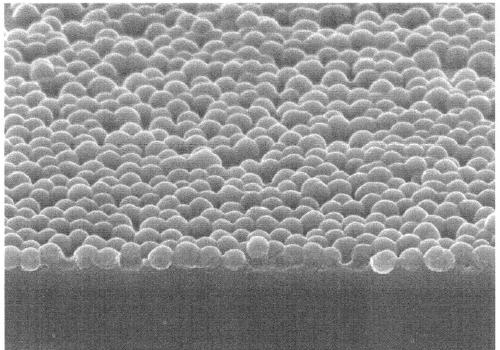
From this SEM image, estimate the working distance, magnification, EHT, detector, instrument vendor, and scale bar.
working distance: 11.7 mm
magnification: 50 K X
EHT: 5 kV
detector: SE(U)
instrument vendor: Hitachi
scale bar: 1000 nm